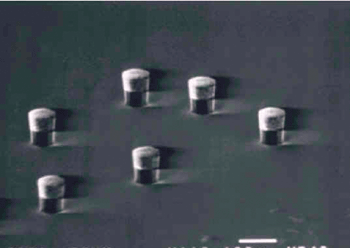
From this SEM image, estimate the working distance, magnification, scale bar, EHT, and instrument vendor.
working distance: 48 mm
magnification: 0.11 K X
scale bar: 100000 nm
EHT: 20 kV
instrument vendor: JEOL